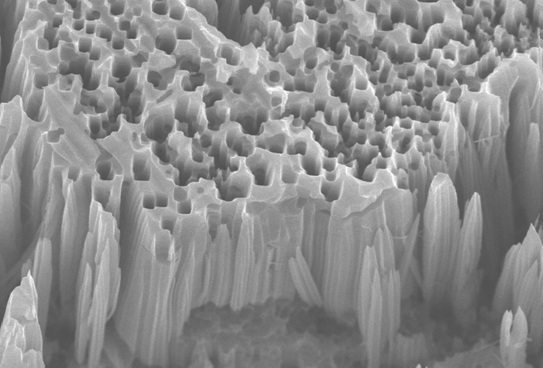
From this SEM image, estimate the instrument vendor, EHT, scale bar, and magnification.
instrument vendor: JEOL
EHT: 15 kV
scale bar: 5000 nm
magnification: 5 K X